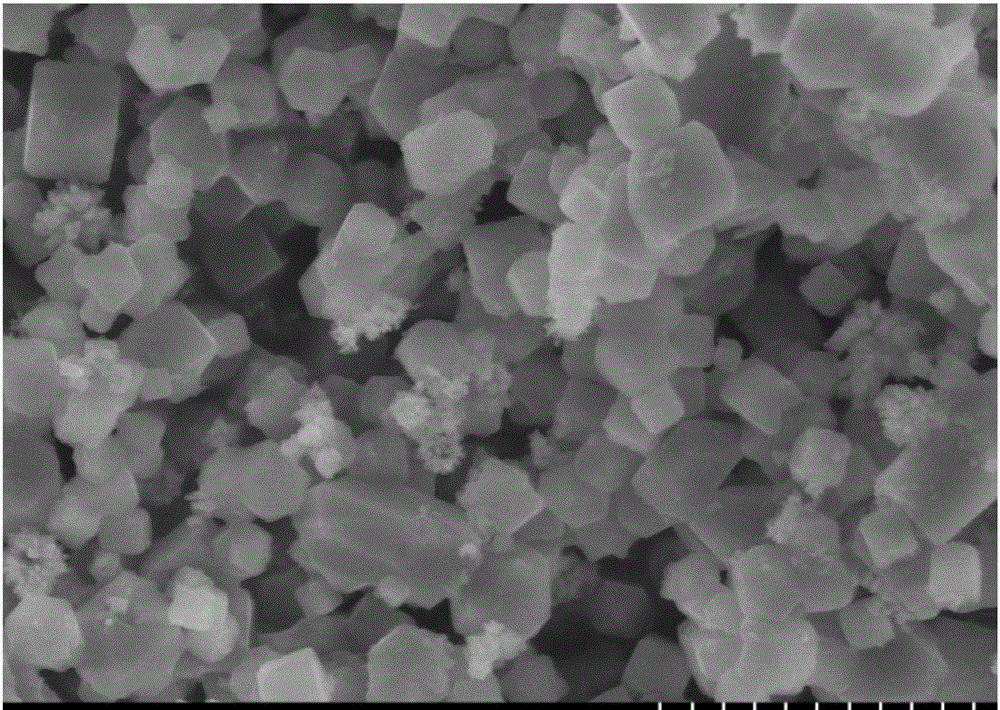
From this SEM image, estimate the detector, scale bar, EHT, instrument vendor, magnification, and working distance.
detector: SE(L)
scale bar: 2000 nm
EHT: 5 kV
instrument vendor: Hitachi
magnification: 20 K X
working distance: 5.6 mm